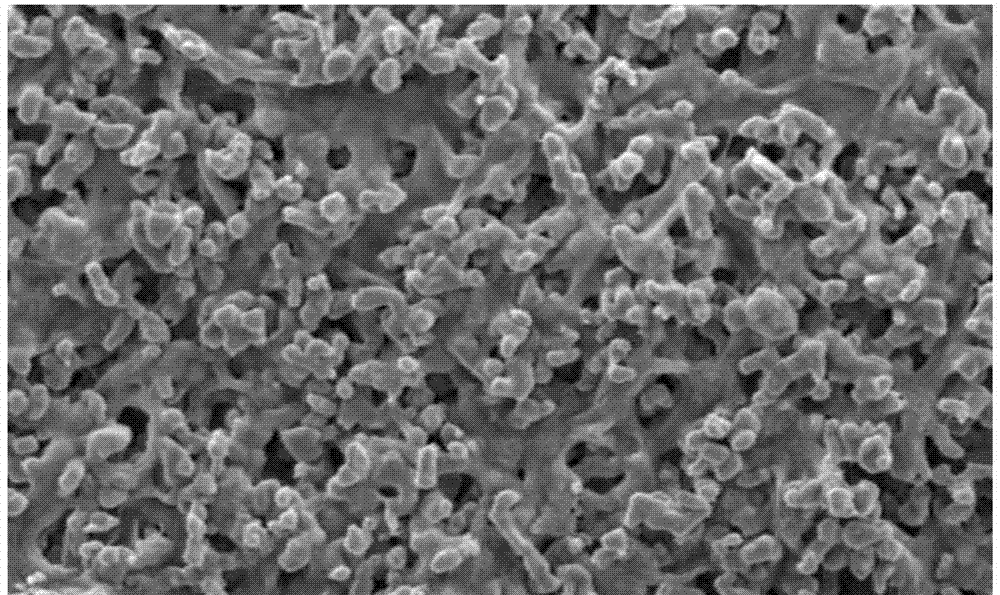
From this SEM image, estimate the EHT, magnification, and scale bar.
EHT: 3.5 kV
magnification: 10 K X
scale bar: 1000 nm